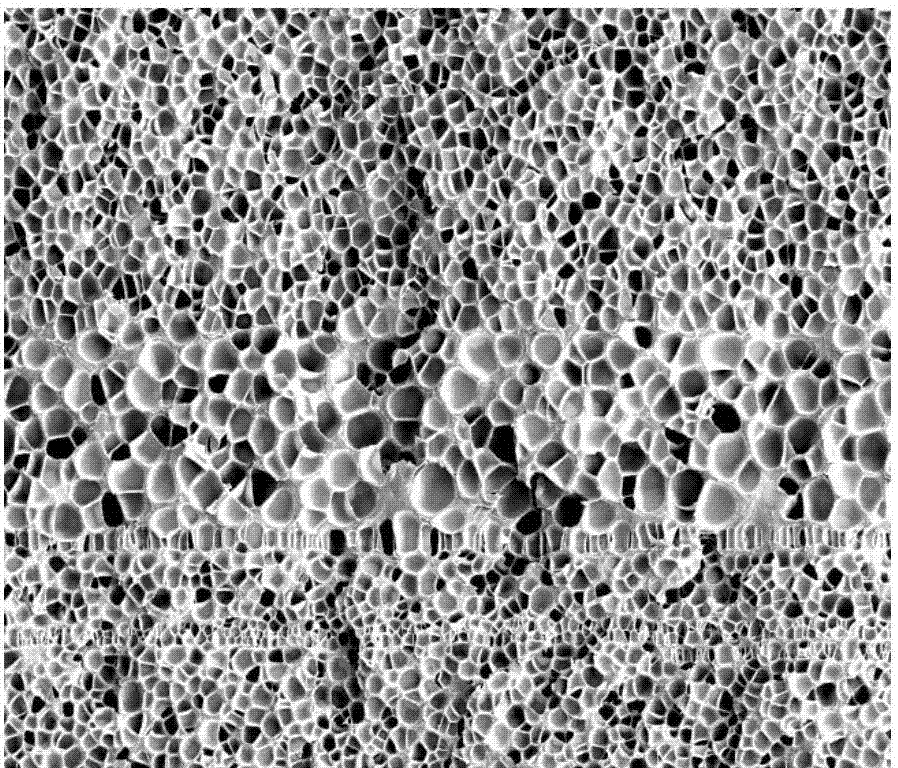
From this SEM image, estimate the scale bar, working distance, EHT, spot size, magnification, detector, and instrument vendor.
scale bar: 100000 nm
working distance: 9.9 mm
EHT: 5 kV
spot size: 4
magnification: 1 K X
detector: ETD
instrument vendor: FEI